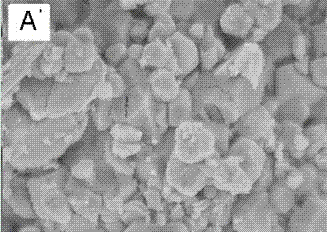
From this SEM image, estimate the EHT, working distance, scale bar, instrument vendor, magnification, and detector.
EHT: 3 kV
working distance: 3 mm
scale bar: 1000 nm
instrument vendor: Hitachi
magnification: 50 K X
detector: SE(UL)